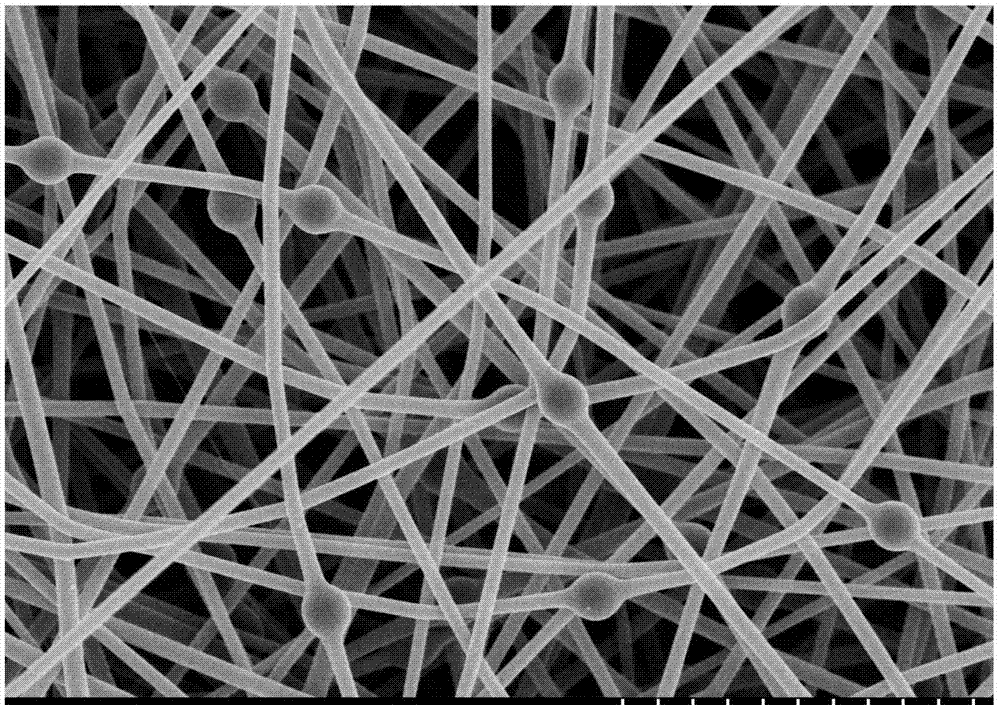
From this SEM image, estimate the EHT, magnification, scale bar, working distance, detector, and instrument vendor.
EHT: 5 kV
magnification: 9 K X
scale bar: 5000 nm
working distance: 8.8 mm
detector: SE(M)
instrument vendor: Hitachi